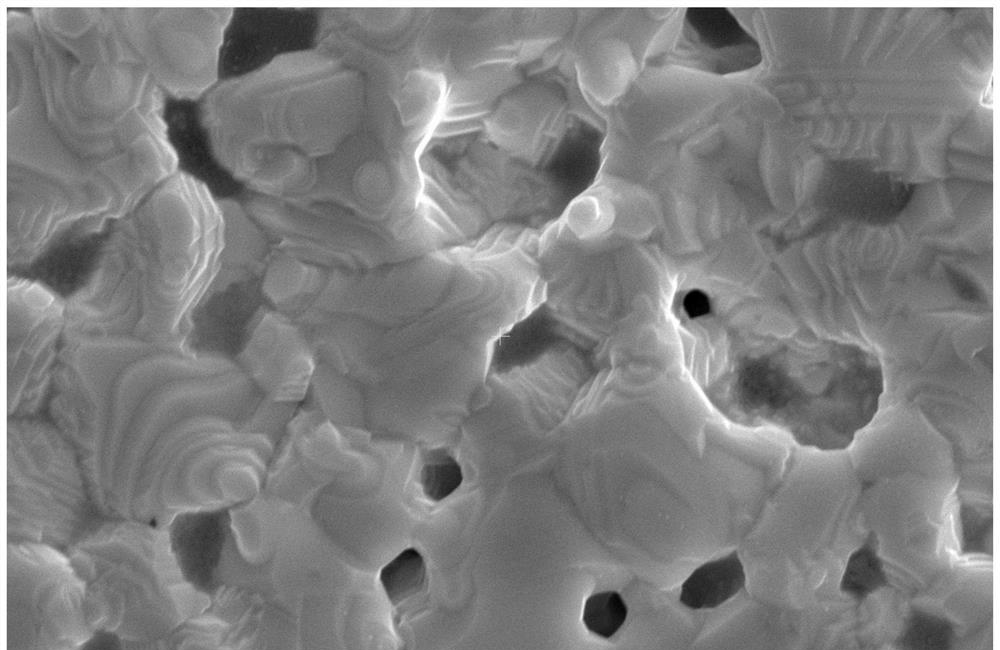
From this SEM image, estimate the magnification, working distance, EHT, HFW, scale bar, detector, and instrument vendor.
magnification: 10 K X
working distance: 10.8192 mm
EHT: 15 kV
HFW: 12.7 µm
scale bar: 2000 nm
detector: ETD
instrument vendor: FEI